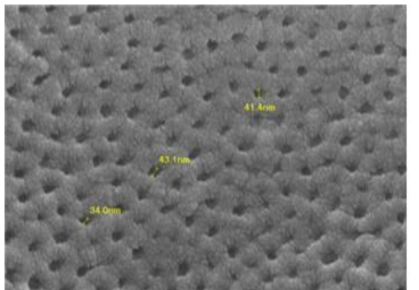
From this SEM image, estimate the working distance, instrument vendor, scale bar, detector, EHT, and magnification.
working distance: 5.2 mm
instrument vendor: JEOL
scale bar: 100 nm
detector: SEI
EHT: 10 kV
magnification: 75 K X